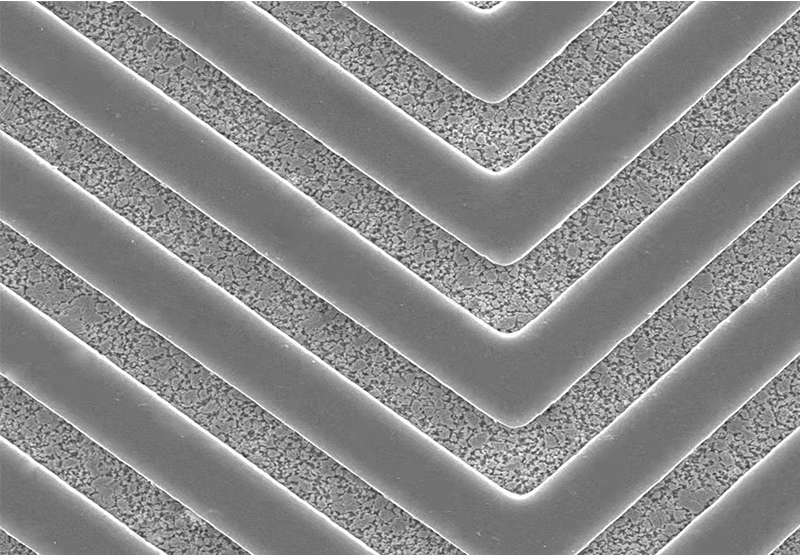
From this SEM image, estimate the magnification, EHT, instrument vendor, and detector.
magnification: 0.5 K X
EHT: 20 kV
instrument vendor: Hitachi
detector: SE